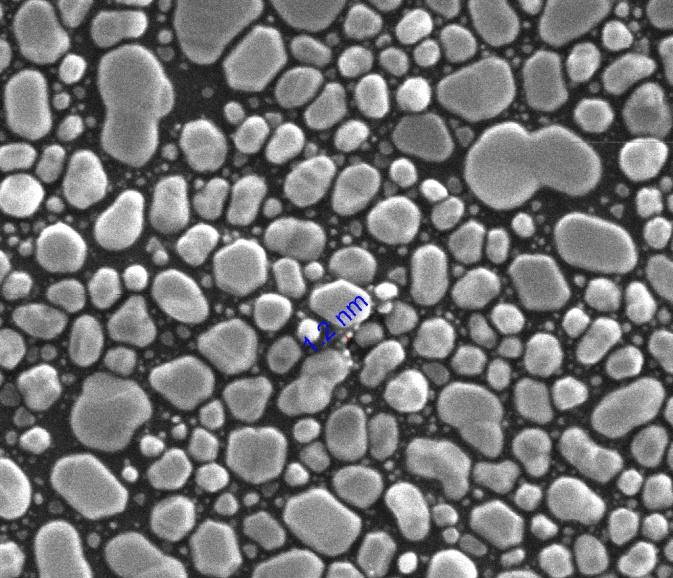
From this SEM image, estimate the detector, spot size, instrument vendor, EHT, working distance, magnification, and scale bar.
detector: ETD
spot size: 3.5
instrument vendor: FEI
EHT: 30 kV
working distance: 4.1 mm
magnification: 200 K X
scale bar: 200 nm